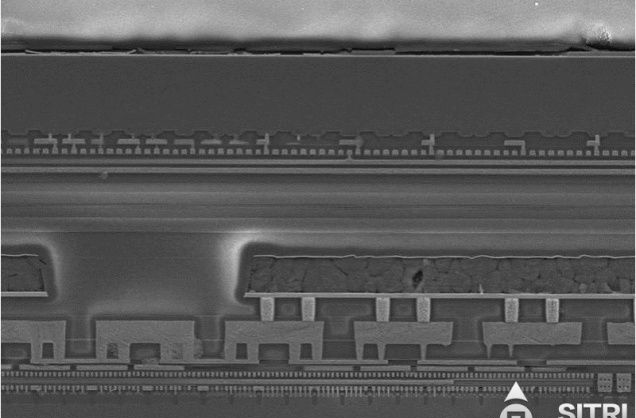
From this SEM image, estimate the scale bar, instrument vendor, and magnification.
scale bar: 1000 nm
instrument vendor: Zeiss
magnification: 13 K X